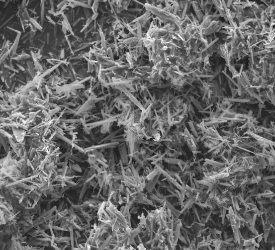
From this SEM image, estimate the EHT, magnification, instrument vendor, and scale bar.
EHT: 15 kV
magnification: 2 K X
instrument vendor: FEI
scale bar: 50000 nm